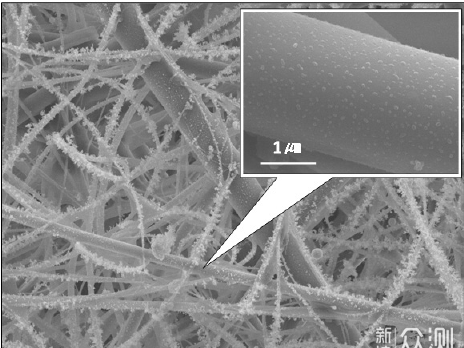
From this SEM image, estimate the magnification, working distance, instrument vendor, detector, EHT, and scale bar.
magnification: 1.5 K X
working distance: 9.9 mm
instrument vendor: JEOL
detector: SEI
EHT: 15 kV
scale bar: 10000 nm